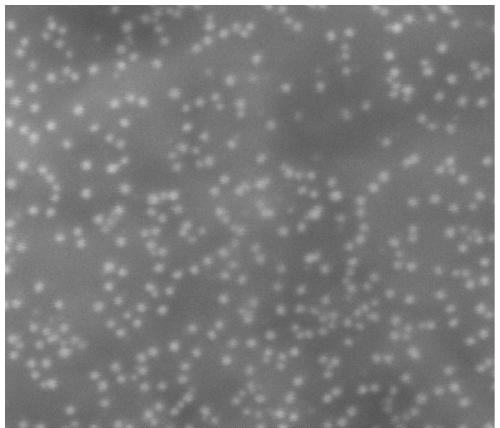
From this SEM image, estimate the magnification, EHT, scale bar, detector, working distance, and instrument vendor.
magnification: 200 K X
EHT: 10 kV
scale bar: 400 nm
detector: ETD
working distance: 10.6 mm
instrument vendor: FEI